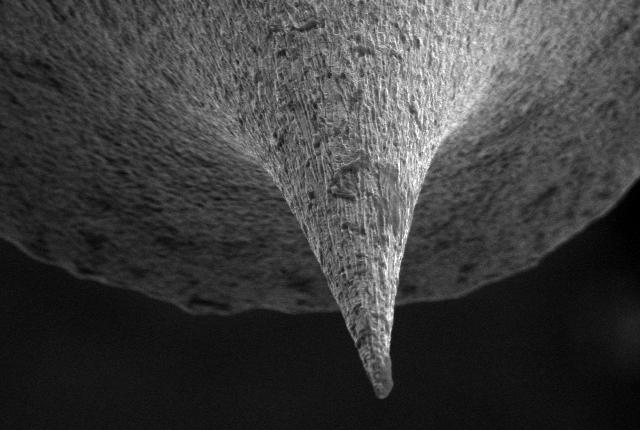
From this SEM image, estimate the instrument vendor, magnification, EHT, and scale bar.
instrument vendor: JEOL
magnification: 1 K X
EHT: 15 kV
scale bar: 10000 nm